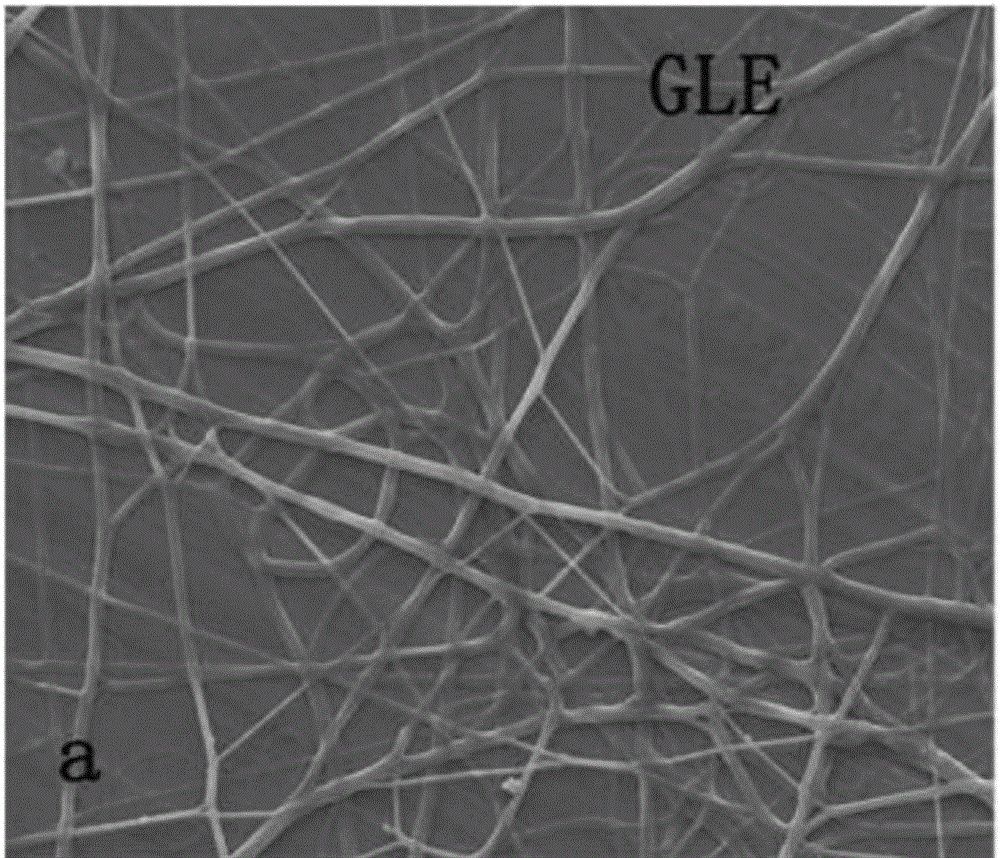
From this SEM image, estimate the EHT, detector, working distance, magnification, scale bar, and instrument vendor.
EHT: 20 kV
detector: ETD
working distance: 12.4 mm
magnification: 1 K X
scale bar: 100000 nm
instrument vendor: FEI